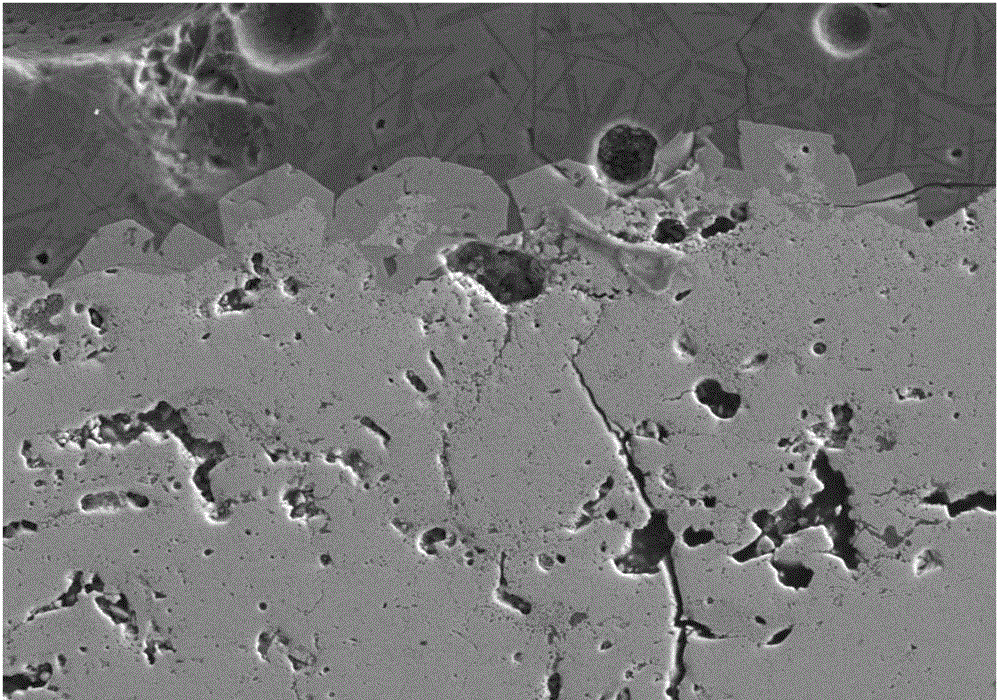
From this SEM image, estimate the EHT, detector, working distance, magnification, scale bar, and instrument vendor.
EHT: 20 kV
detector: SE(M,LA100)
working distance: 15 mm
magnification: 0.5 K X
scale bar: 100000 nm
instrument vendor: Hitachi